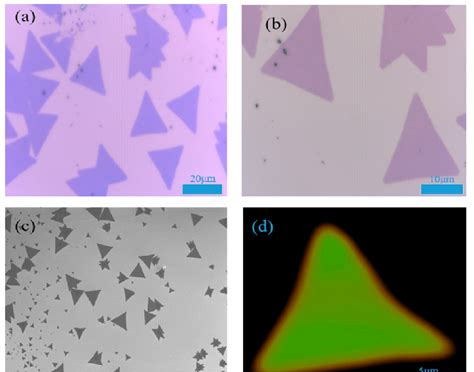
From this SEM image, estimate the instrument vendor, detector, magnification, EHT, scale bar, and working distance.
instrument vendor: JEOL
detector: SEI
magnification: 0.5 K X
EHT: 5 kV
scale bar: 10000 nm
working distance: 8.1 mm